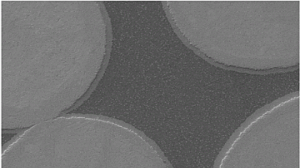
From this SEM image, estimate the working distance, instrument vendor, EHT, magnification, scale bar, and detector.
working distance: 9 mm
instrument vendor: Zeiss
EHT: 20 kV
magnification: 0.5 K X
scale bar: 10000 nm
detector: SE1